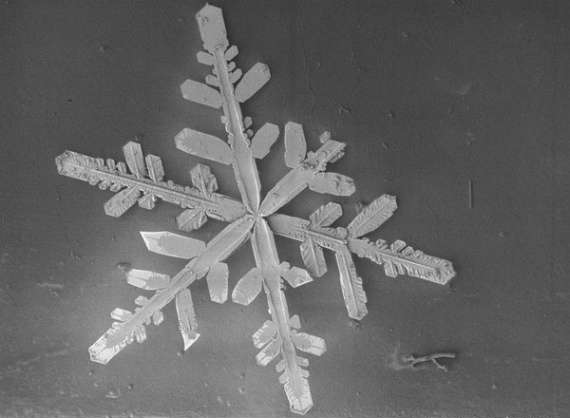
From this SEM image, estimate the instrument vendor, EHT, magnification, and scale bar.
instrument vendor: JEOL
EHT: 2 kV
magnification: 0.04 K X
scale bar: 750000 nm